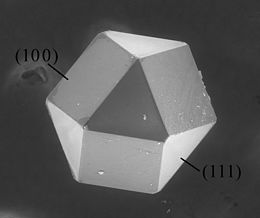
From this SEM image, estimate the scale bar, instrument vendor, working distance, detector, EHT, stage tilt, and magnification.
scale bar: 200000 nm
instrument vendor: FEI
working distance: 13.1 mm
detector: LFD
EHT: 20 kV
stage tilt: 0°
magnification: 0.4 K X